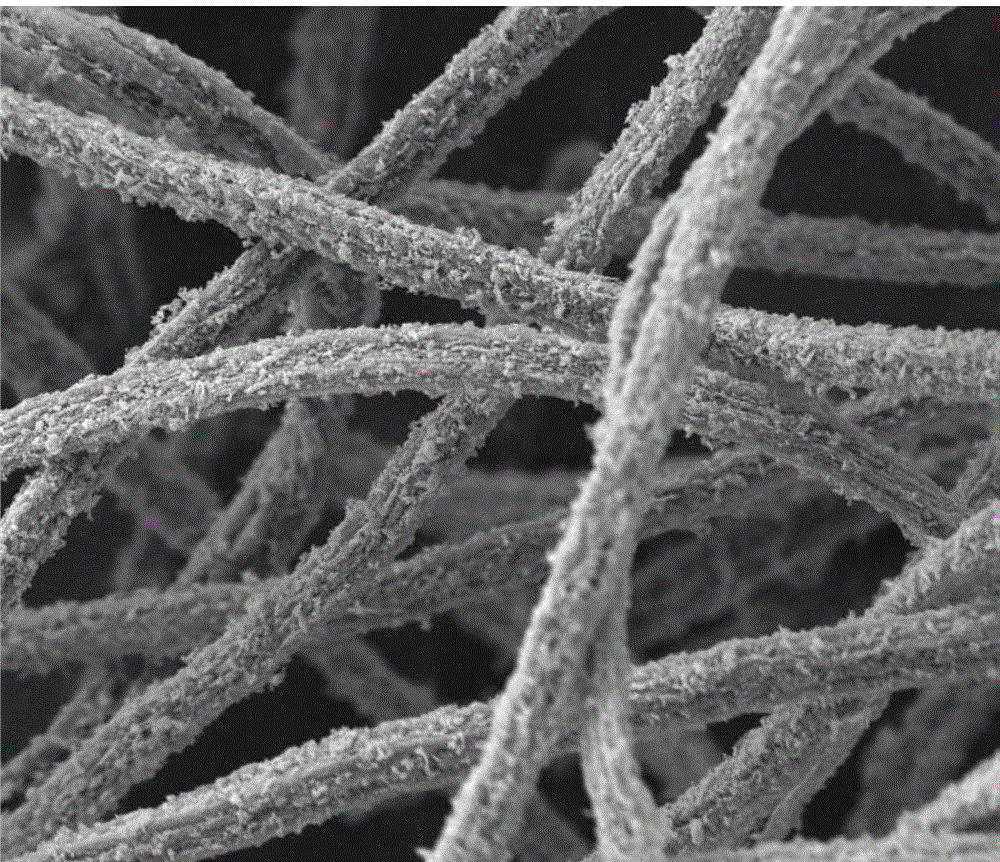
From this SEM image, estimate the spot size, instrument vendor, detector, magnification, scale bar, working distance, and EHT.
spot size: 5.5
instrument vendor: FEI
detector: ETD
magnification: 1 K X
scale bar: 20000 nm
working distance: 9.5 mm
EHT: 15 kV